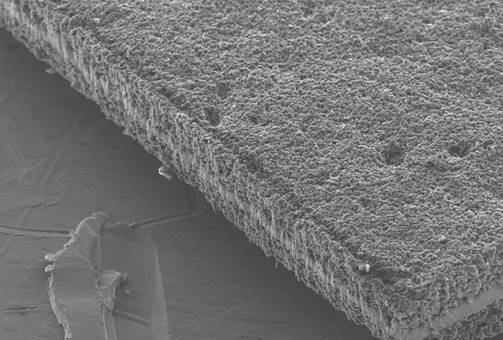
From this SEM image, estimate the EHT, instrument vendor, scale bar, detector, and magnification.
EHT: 15 kV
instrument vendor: JEOL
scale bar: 200000 nm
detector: SEI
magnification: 0.1 K X